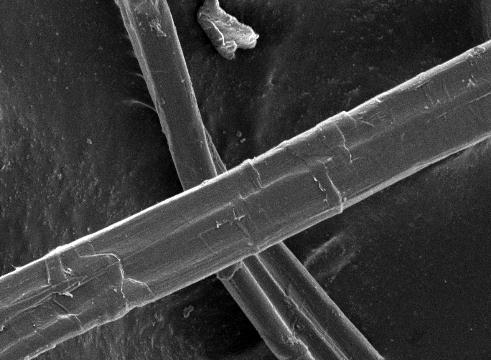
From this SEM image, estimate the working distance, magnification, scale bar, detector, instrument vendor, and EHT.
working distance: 8 mm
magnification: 0.85 K X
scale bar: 10000 nm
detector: SEI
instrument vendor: JEOL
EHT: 5 kV